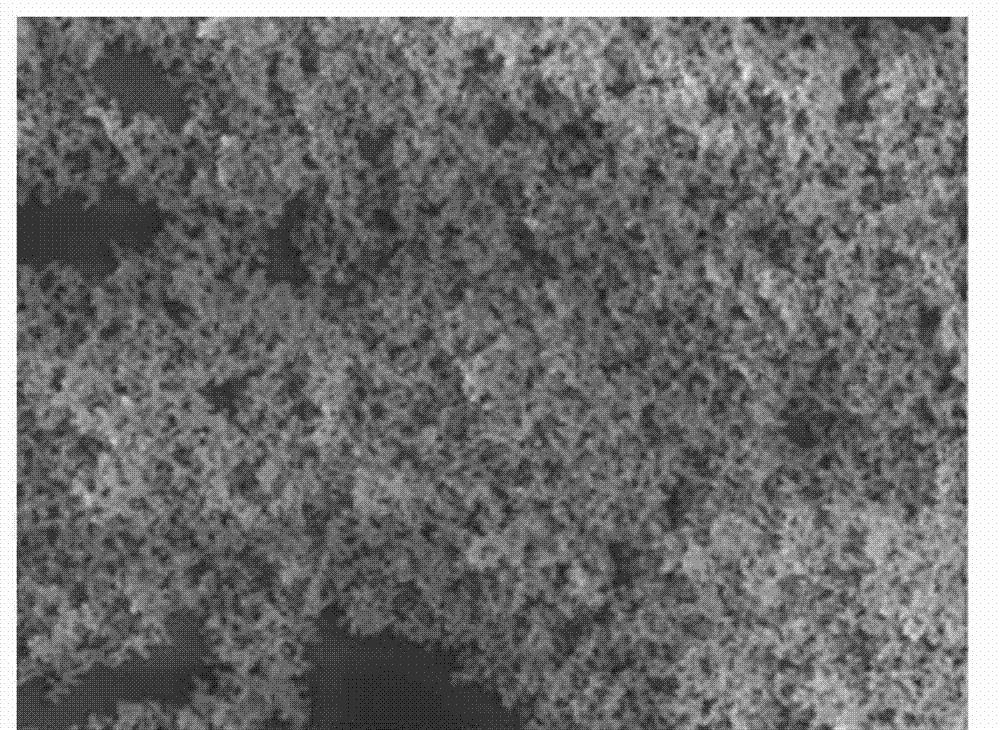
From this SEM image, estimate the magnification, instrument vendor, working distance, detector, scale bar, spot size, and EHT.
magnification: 5 K X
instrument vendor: FEI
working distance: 8.9 mm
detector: ETD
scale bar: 5000 nm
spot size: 3.5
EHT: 20 kV